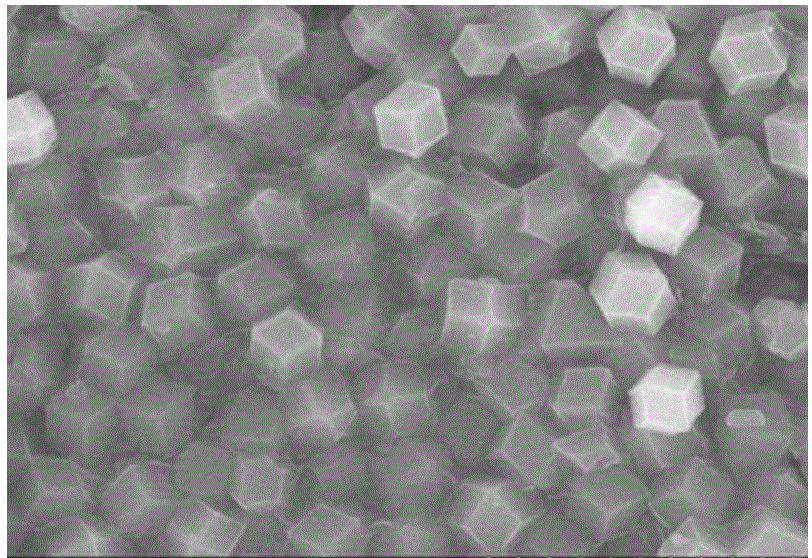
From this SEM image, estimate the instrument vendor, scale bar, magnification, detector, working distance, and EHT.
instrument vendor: Hitachi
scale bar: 4000 nm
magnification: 12 K X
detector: SE(M,LA100)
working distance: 9.3 mm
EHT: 15 kV